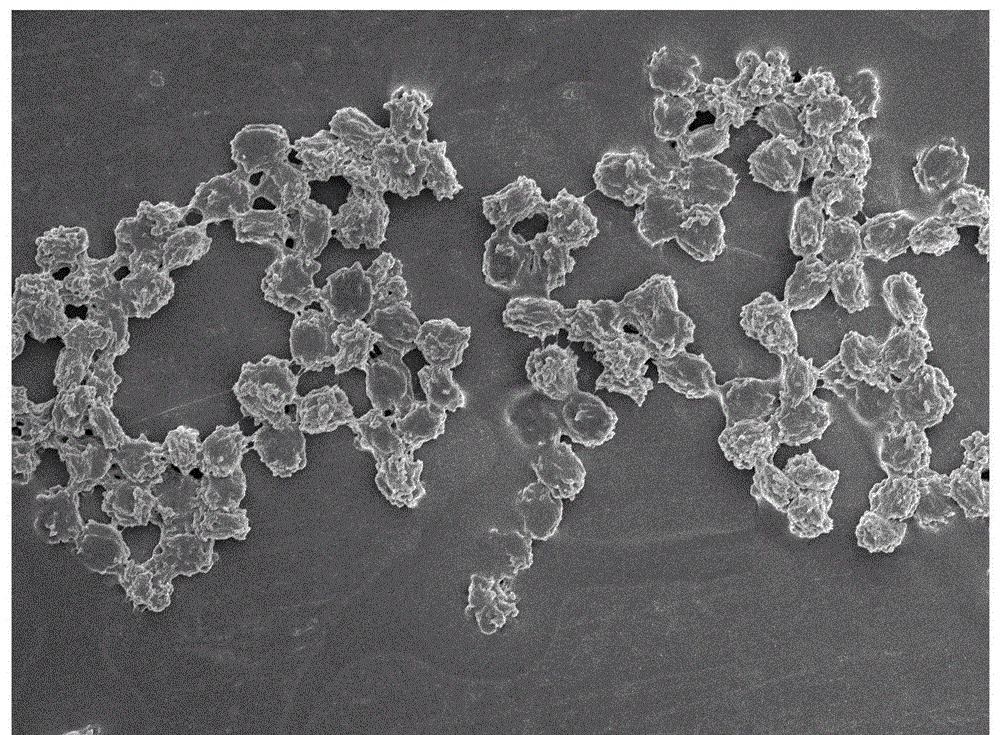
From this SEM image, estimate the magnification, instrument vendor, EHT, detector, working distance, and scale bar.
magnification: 2 K X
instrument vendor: JEOL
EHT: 3 kV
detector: SEI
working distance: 8.2 mm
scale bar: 10000 nm